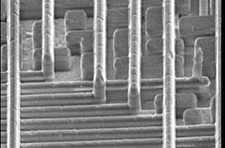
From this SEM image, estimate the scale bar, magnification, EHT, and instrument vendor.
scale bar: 2000 nm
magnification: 9.5 K X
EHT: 5 kV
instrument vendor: JEOL